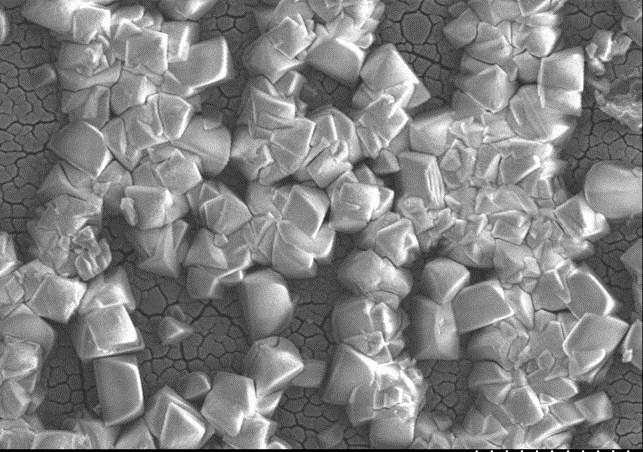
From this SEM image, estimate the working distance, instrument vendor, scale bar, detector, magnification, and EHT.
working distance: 9.8 mm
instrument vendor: Hitachi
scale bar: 10000 nm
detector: SE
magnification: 3 K X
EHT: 5 kV